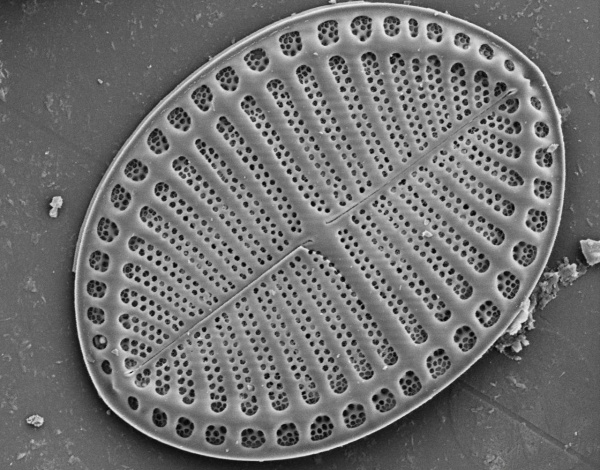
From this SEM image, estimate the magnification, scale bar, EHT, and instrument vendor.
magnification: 2 K X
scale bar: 10000 nm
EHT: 5 kV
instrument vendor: JEOL